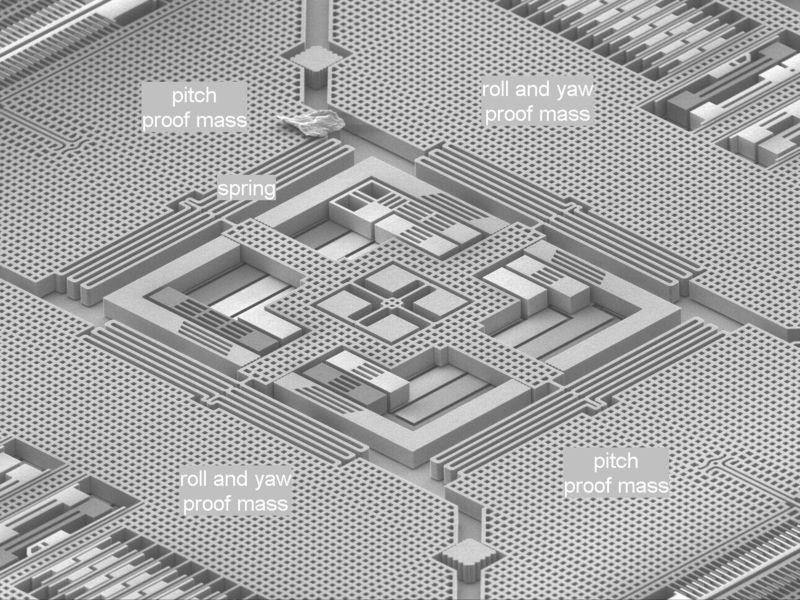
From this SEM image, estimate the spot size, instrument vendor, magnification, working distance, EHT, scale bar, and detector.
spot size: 3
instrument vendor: FEI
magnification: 0.15 K X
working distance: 15.5 mm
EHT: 10 kV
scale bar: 200000 nm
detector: SE